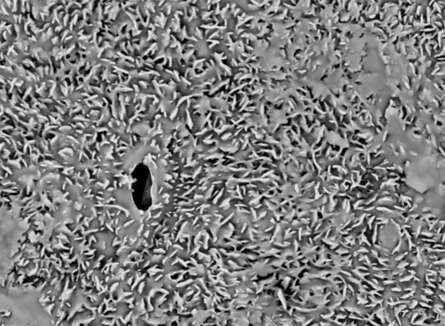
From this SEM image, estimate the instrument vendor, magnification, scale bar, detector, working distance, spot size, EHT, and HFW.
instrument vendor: FEI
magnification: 2.5 K X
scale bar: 10000 nm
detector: BSE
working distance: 10.7 mm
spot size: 4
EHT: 10 kV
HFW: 49.6 µm